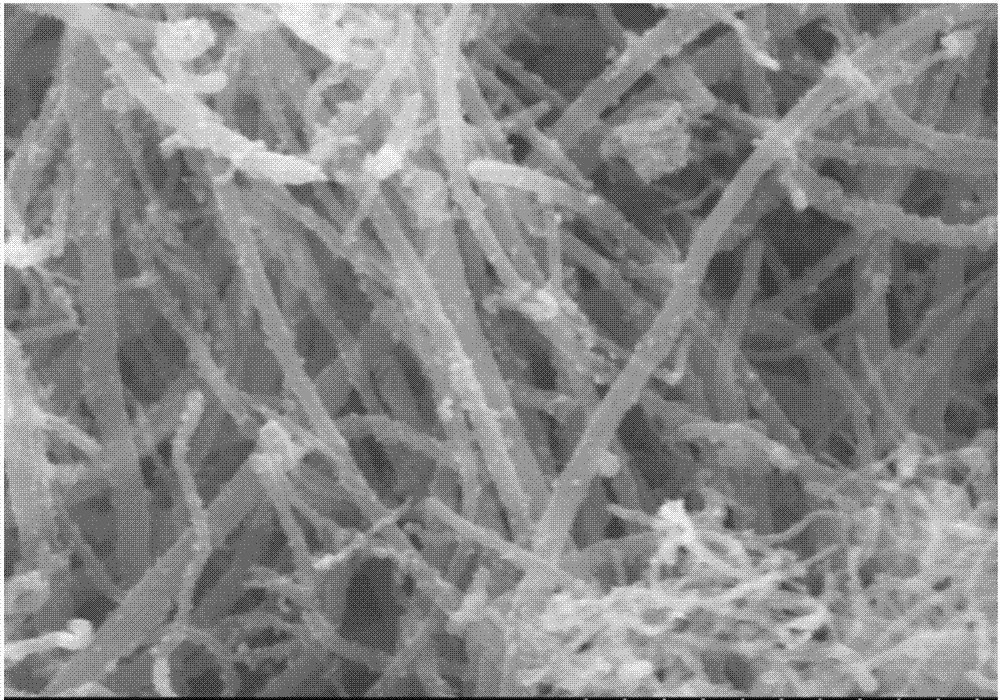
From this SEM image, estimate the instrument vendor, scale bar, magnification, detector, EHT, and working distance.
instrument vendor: Hitachi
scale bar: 1000 nm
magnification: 50 K X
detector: SE(U)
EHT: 5 kV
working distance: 9.4 mm